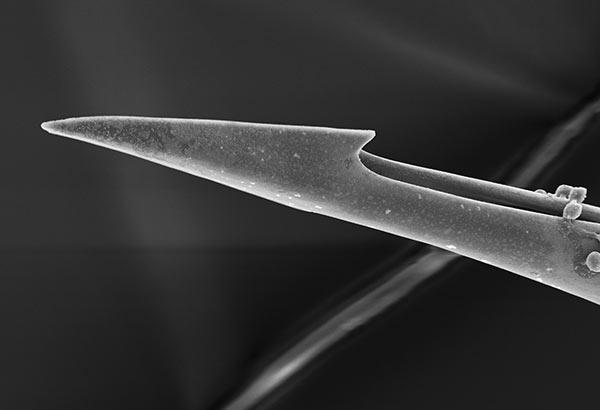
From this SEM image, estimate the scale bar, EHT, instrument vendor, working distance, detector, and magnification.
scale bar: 50000 nm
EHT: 15 kV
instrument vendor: Hitachi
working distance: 14.9 mm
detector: SE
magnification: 0.8 K X